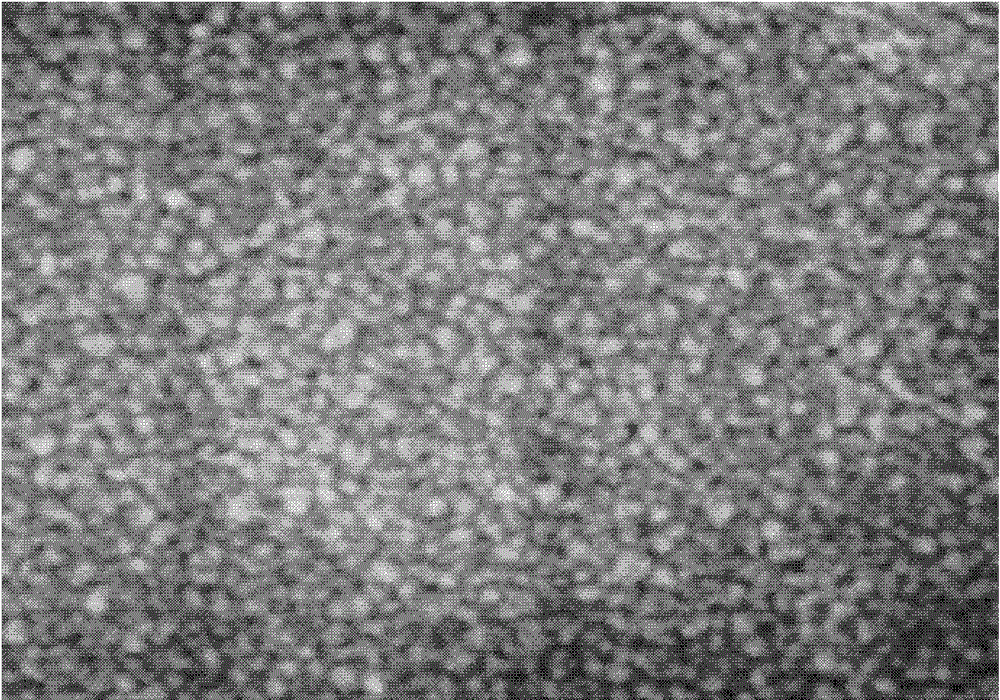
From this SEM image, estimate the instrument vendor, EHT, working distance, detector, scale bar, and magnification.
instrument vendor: Hitachi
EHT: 1 kV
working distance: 10.9 mm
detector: SE(U)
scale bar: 500 nm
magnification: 80 K X